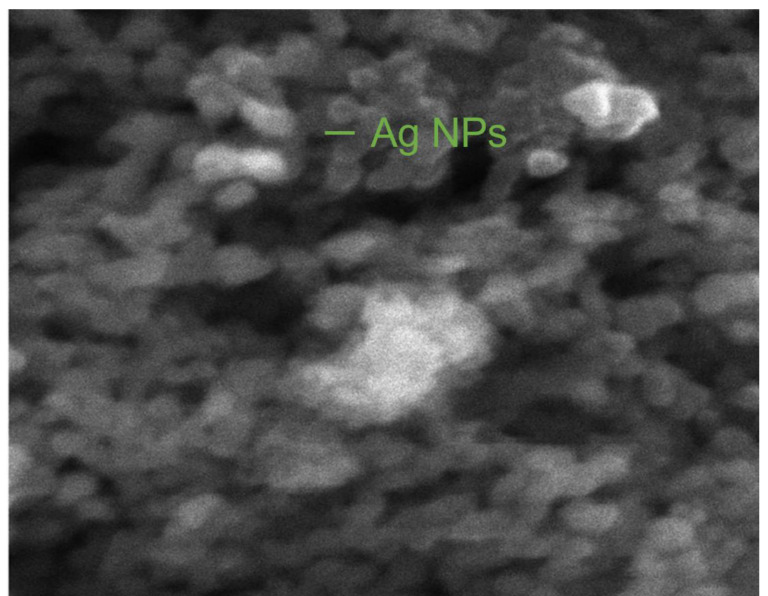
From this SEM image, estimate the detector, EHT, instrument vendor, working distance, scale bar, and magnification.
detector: SE + InBeam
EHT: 10 kV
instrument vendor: Tescan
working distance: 6.39 mm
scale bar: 500 nm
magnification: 100 K X